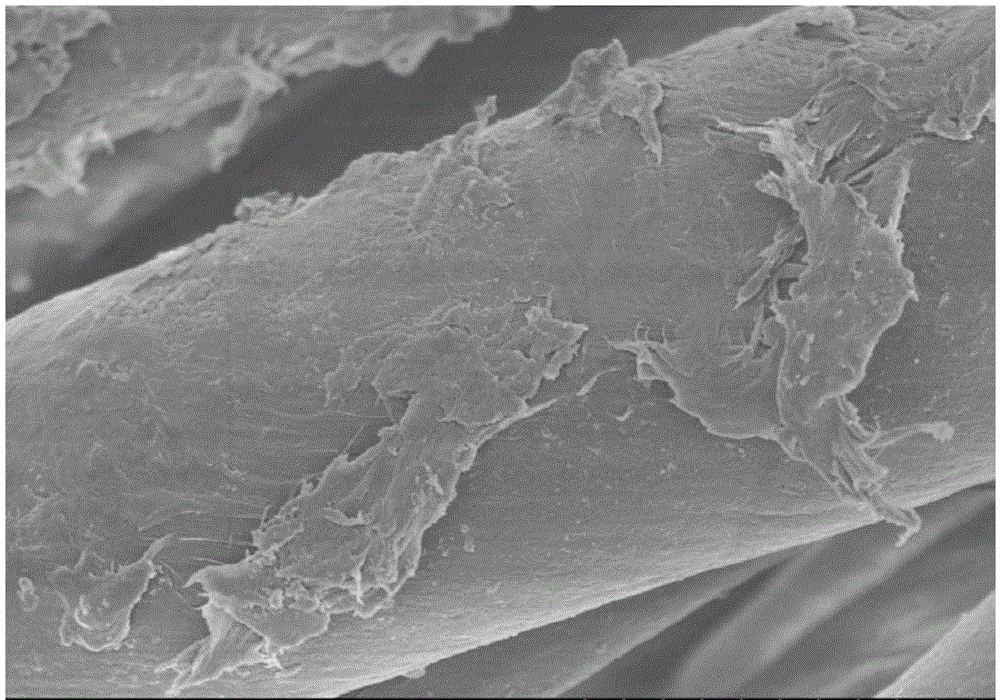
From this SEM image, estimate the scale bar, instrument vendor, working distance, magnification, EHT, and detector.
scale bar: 10000 nm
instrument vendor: Hitachi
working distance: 8.4 mm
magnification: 5 K X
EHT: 3 kV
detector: SE(M)